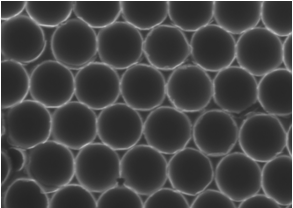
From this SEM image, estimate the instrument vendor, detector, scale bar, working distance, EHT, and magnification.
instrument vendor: JEOL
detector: SEI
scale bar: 1000 nm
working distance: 12 mm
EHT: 15 kV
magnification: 20 K X